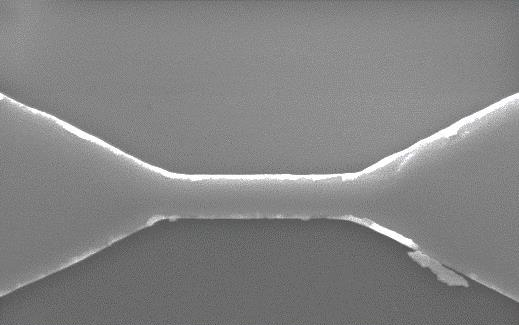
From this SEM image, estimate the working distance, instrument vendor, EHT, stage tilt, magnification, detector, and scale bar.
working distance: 13 mm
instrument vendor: Zeiss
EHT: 5 kV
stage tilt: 0°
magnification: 60 K X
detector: SE2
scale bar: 200 nm